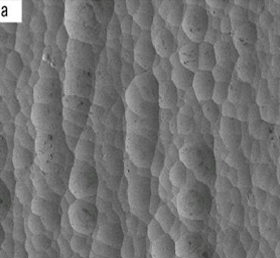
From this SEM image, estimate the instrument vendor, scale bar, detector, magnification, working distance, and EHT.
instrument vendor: JEOL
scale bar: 10000 nm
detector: LEI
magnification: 0.5 K X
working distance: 8.3 mm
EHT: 5 kV